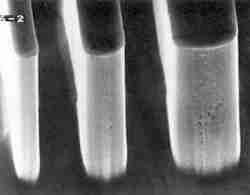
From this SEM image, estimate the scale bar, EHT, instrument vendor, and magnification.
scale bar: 1000 nm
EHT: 15 kV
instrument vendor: JEOL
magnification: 10 K X